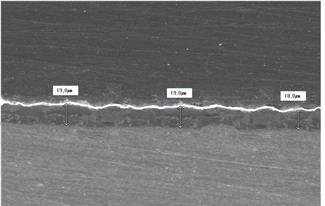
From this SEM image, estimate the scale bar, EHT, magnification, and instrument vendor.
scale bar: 50000 nm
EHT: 15 kV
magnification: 0.4 K X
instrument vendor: JEOL